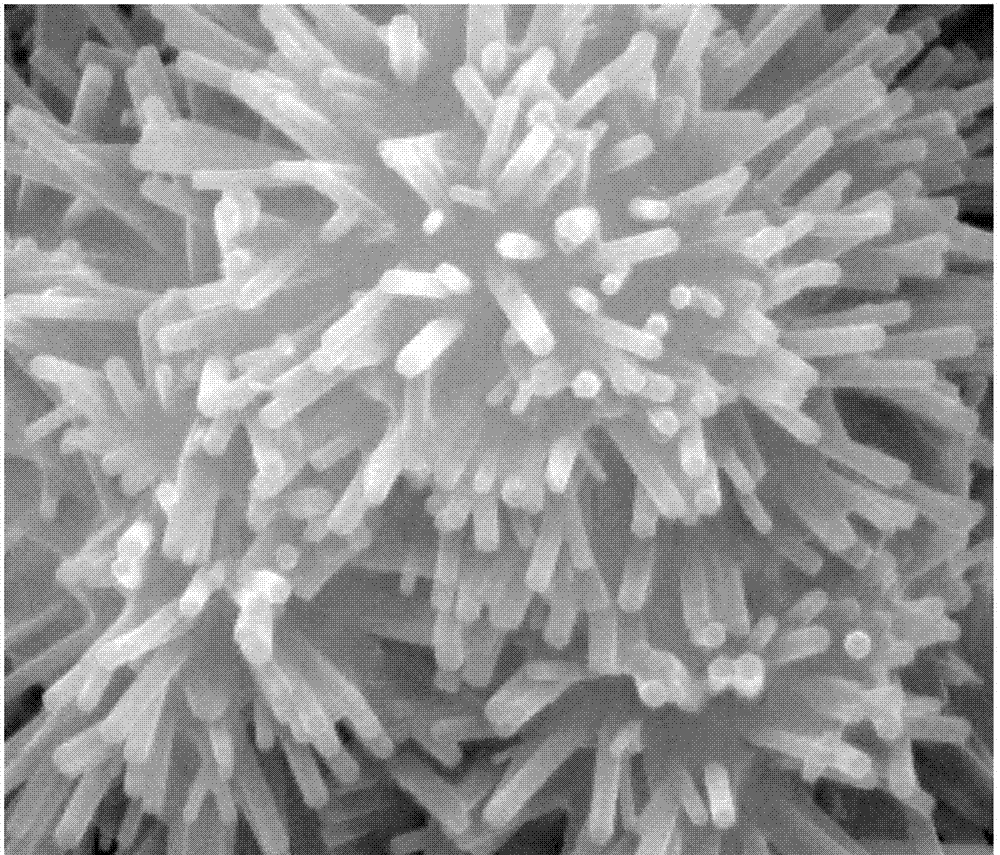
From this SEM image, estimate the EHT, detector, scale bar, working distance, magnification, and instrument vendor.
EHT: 30 kV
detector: SE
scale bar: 2000 nm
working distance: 11.9 mm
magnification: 50 K X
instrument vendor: FEI